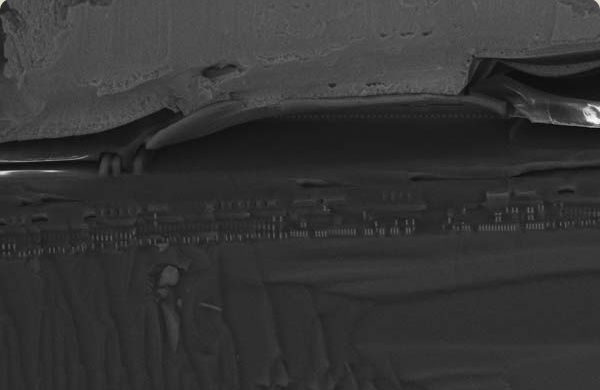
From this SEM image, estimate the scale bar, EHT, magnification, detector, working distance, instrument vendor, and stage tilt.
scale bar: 10000 nm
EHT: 15 kV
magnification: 3.16 K X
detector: InLens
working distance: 4 mm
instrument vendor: Zeiss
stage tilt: -15°